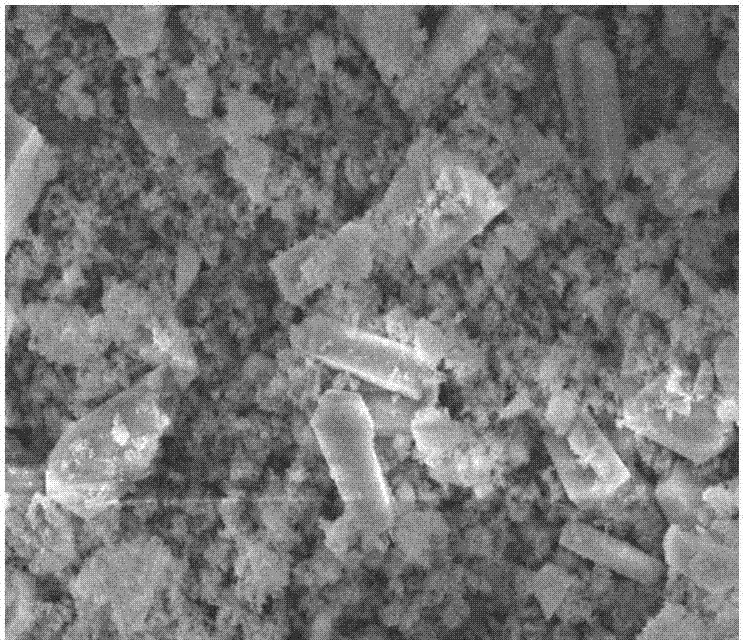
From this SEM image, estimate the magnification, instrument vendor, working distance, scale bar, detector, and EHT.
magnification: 5 K X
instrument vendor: JEOL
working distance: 8.6 mm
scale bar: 1000 nm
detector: SEI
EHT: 8 kV